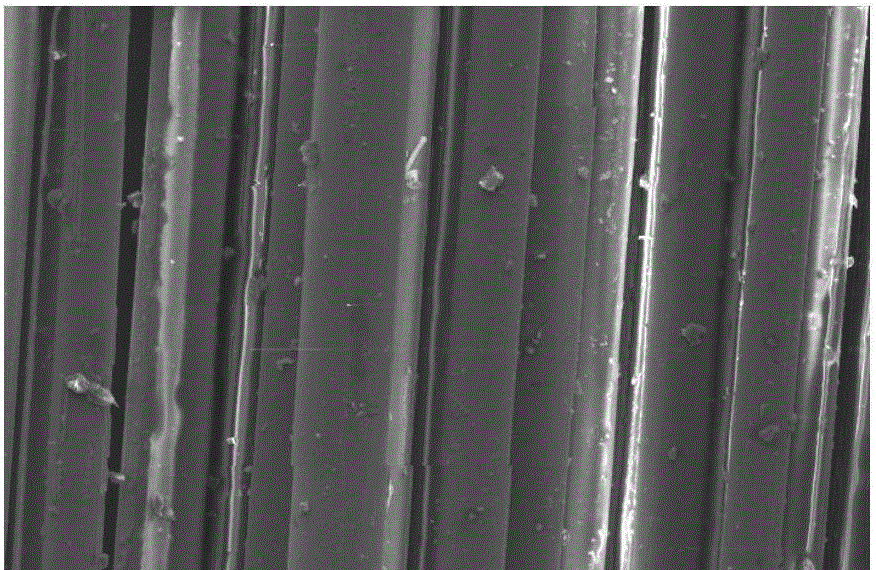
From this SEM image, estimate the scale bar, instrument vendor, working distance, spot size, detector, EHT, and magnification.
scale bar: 50000 nm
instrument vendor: FEI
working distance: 8.1 mm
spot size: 3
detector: SE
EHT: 10 kV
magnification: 0.5 K X